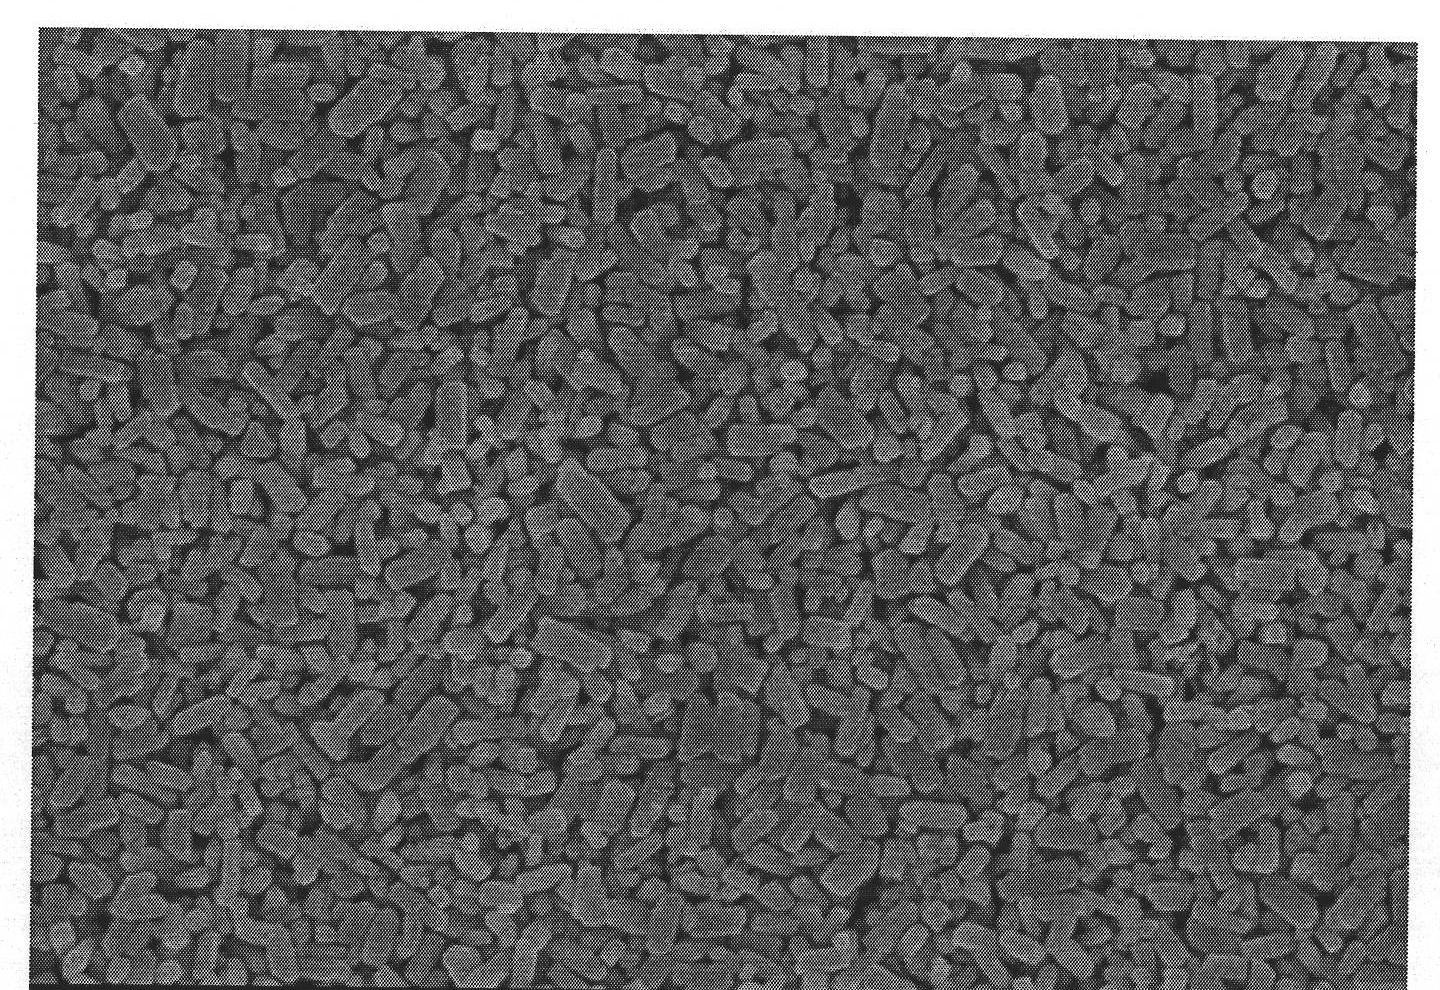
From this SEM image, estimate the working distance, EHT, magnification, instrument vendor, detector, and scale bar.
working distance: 7.8 mm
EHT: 4 kV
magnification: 25 K X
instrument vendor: Hitachi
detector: SE(M)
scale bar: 2000 nm